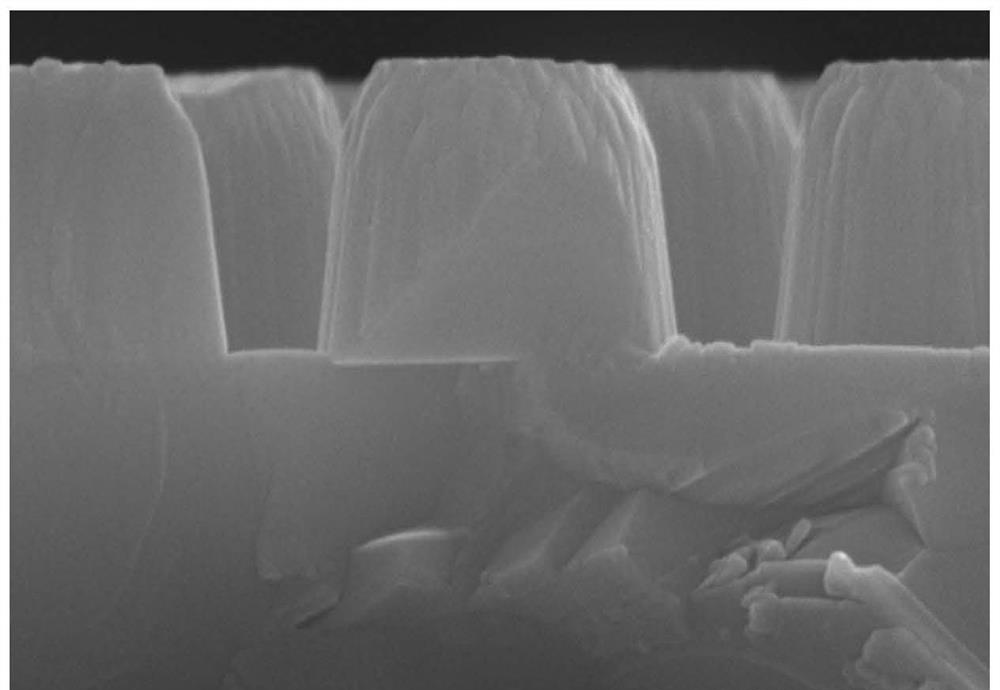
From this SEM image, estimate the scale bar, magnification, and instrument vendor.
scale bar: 2000 nm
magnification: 20 K X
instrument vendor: Hitachi